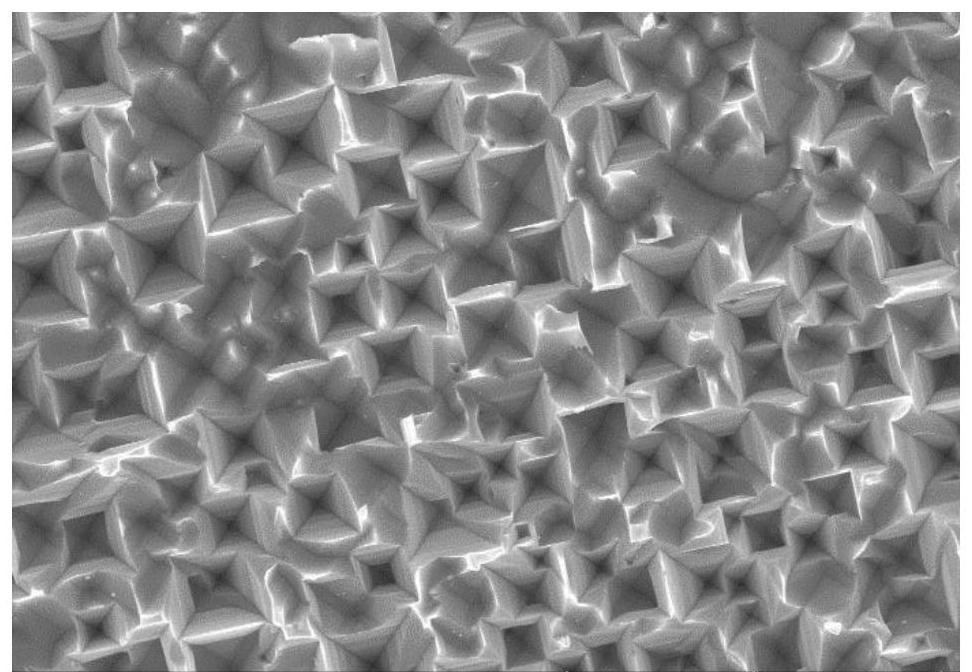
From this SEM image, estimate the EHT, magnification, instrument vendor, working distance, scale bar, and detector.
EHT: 10 kV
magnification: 5 K X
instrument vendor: Hitachi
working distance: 9.6 mm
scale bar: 10000 nm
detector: SE(U)